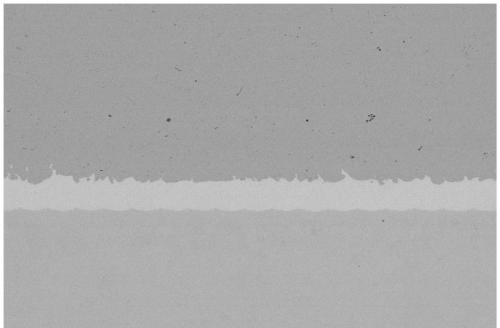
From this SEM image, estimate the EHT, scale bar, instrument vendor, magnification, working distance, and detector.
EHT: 10 kV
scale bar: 100000 nm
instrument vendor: Zeiss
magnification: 0.032 K X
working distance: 9 mm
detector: NTS BSD